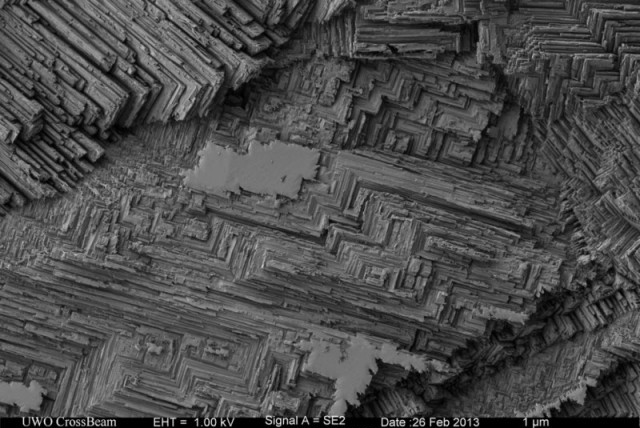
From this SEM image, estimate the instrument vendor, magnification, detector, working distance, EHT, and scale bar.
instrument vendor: Zeiss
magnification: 5 K X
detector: SE2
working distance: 4.8 mm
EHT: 1 kV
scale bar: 1000 nm